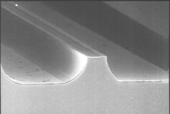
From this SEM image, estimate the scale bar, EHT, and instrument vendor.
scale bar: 10000 nm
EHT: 20 kV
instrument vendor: JEOL